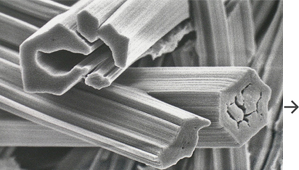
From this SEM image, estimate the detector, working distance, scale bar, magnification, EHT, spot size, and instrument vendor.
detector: SE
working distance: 4.7 mm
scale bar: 2000 nm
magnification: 12 K X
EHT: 5 kV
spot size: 2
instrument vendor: FEI